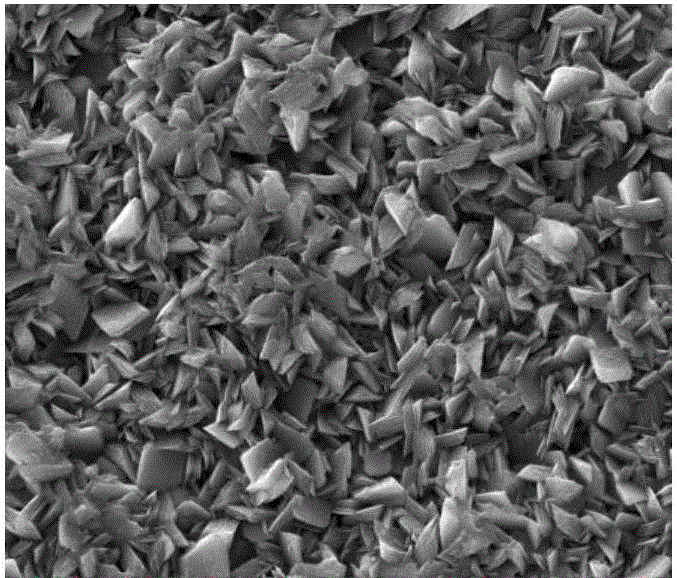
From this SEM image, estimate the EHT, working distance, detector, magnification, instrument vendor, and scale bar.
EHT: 15 kV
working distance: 5.3 mm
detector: ETD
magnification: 8 K X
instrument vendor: FEI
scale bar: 10000 nm